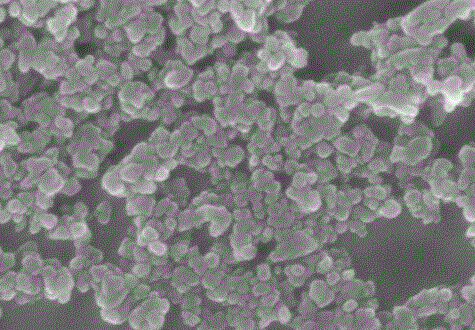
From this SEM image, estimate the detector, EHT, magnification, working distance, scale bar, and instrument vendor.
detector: SE(U)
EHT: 20 kV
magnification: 100 K X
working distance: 8.6 mm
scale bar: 500 nm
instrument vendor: Hitachi